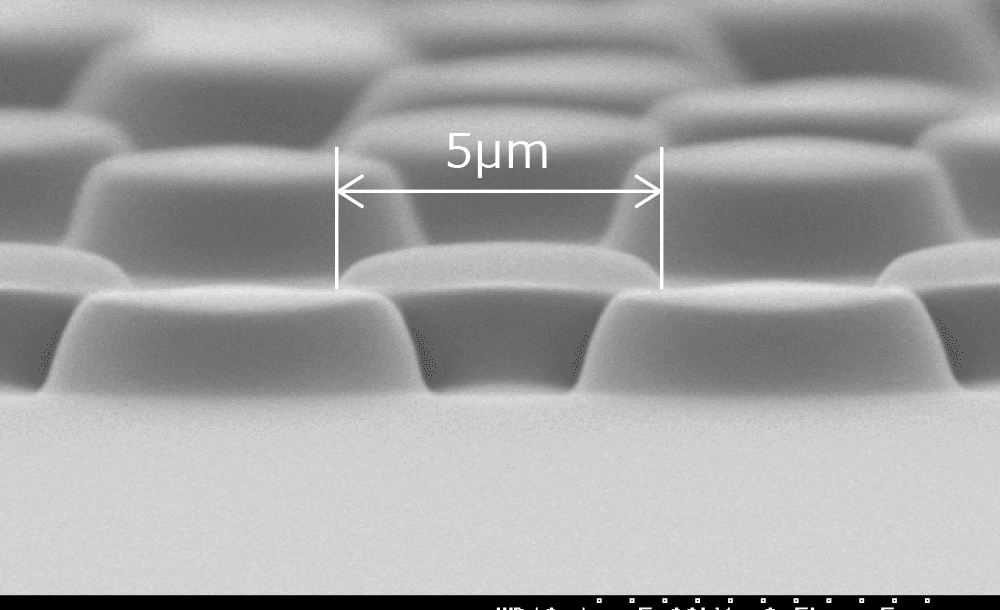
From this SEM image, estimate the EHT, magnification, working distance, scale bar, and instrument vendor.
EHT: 5 kV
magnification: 8.5 K X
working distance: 10.1 mm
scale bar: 5000 nm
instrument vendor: Hitachi